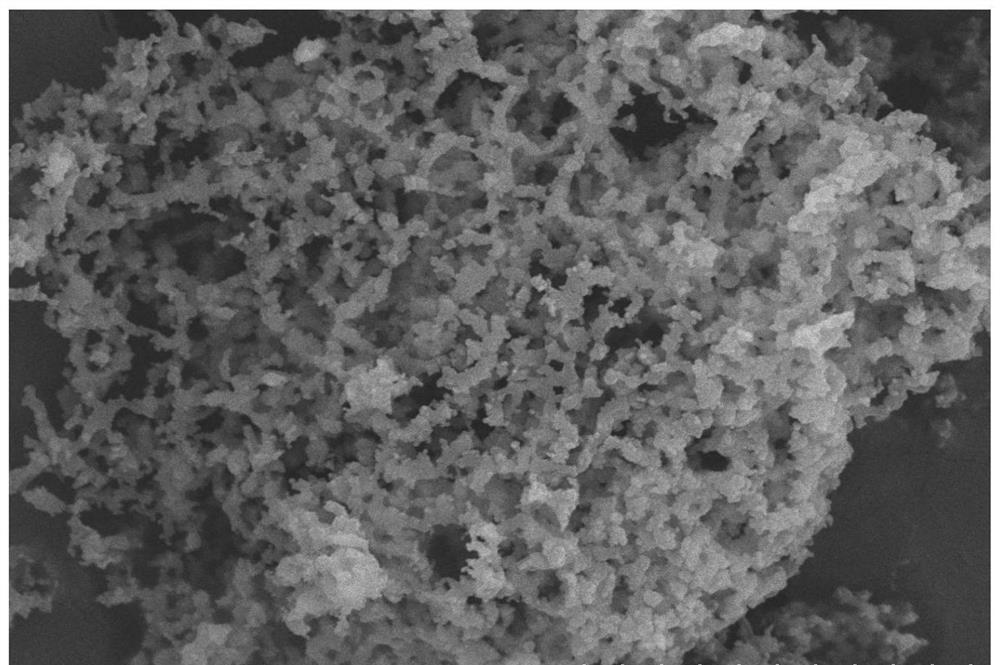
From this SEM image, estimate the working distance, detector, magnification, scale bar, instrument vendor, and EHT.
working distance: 5.5 mm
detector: SE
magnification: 10 K X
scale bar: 5000 nm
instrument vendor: Hitachi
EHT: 15 kV